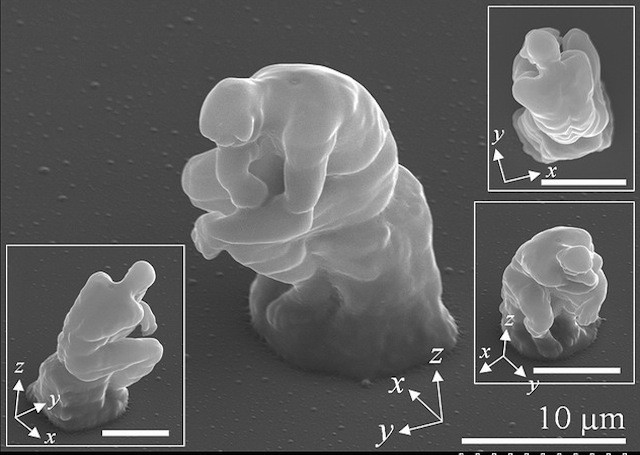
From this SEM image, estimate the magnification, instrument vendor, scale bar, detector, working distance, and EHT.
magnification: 3 K X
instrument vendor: Hitachi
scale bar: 10000 nm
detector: SE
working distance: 10.8 mm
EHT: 15 kV